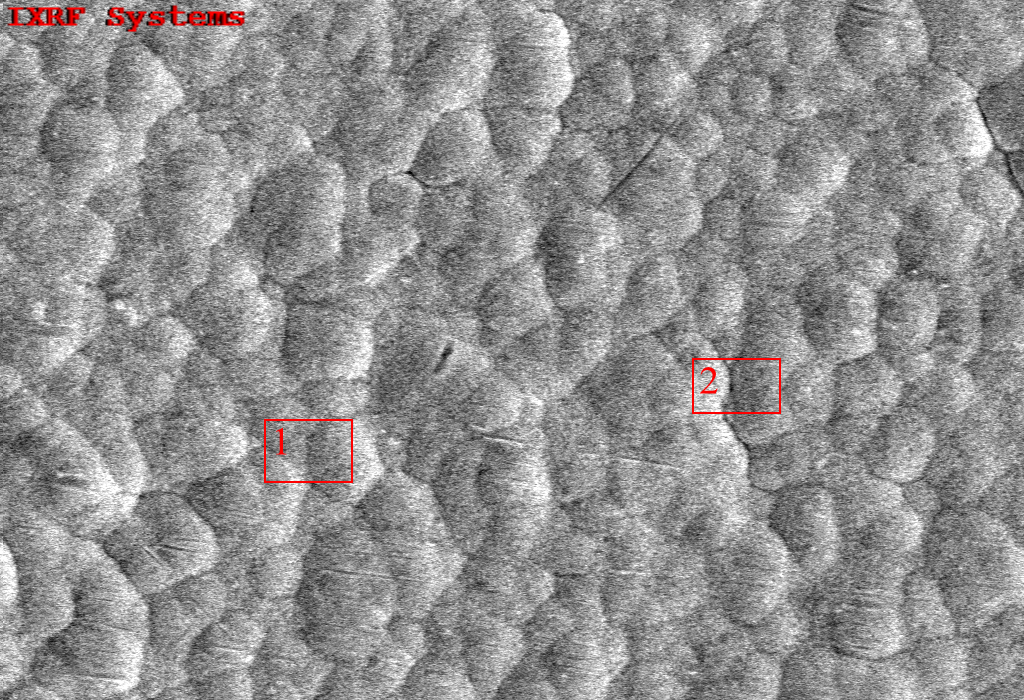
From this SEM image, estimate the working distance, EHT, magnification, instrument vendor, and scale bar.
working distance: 10.1 mm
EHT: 15 kV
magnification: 3 K X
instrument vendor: other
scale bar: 10000 nm